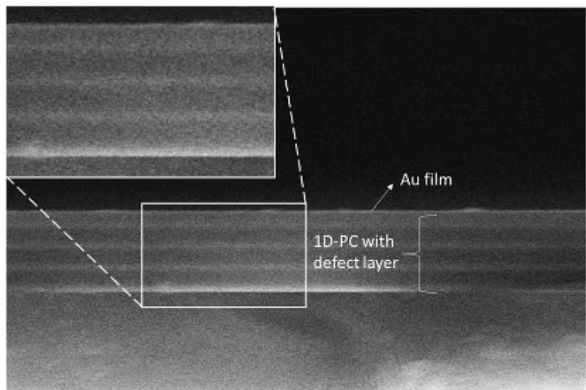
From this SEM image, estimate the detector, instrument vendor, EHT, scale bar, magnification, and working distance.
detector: SE1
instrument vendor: Zeiss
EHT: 20 kV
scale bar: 2000 nm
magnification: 20 K X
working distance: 10 mm